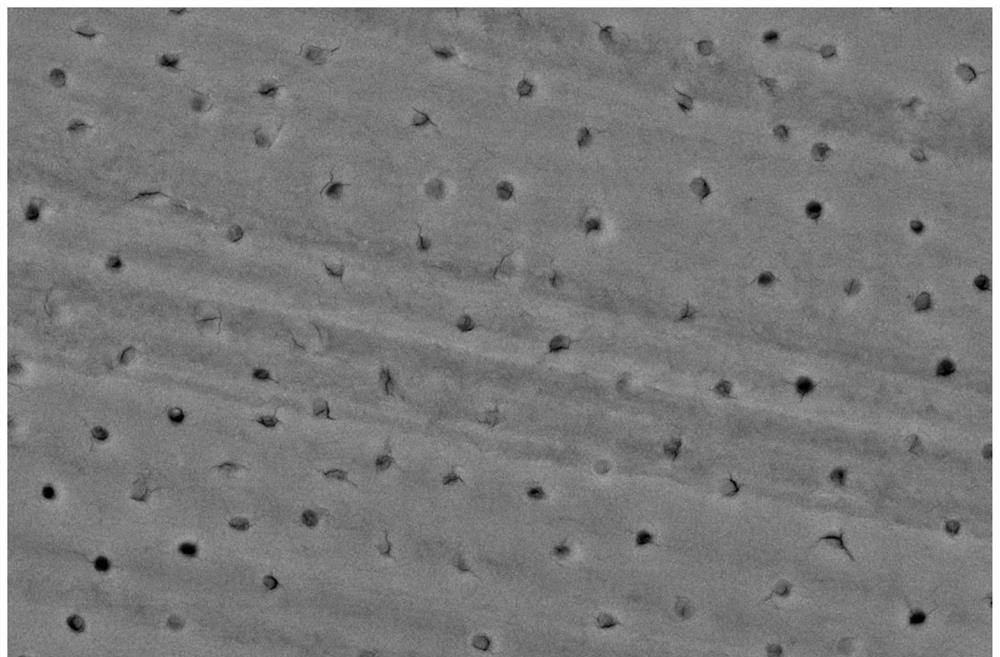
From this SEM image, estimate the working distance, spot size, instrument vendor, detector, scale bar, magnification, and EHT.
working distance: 11.1 mm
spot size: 3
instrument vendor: FEI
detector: Z Cont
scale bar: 10000 nm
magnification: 3 K X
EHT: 20 kV